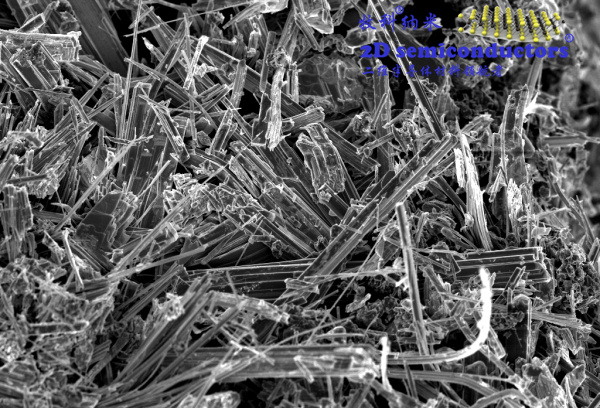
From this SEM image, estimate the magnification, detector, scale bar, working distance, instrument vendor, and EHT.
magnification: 2 K X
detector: InLens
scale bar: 2000 nm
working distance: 7 mm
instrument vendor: Zeiss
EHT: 5 kV